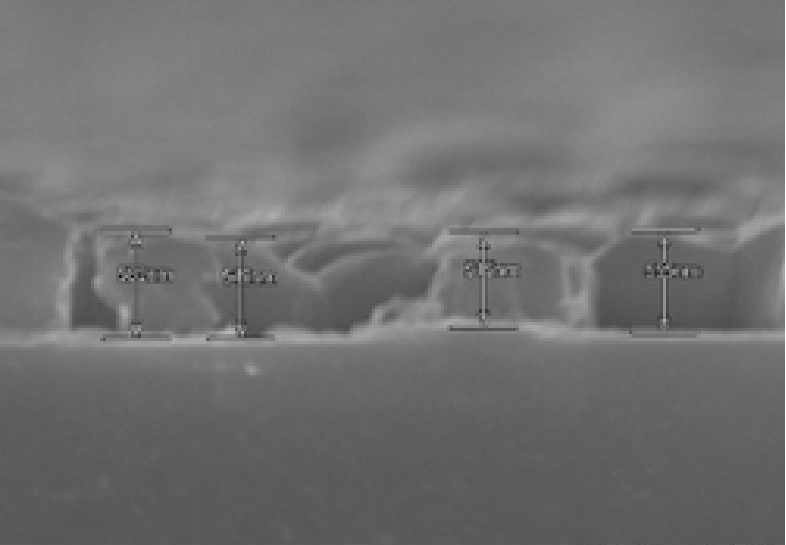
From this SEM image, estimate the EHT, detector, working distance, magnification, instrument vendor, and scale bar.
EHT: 15 kV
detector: SE(U)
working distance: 14 mm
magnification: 30 K X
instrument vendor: Hitachi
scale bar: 1000 nm